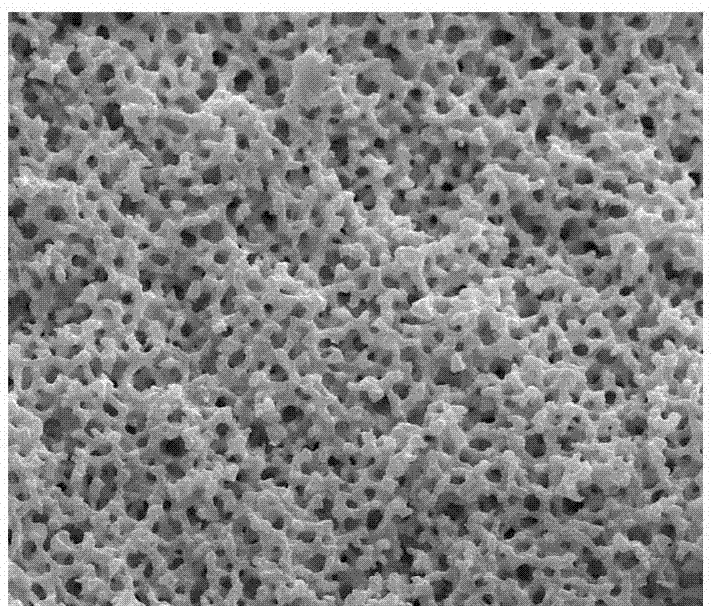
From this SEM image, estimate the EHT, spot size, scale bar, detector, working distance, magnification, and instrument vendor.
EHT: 20 kV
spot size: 3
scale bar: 20000 nm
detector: ETD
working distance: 10.8 mm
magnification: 5 K X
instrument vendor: FEI